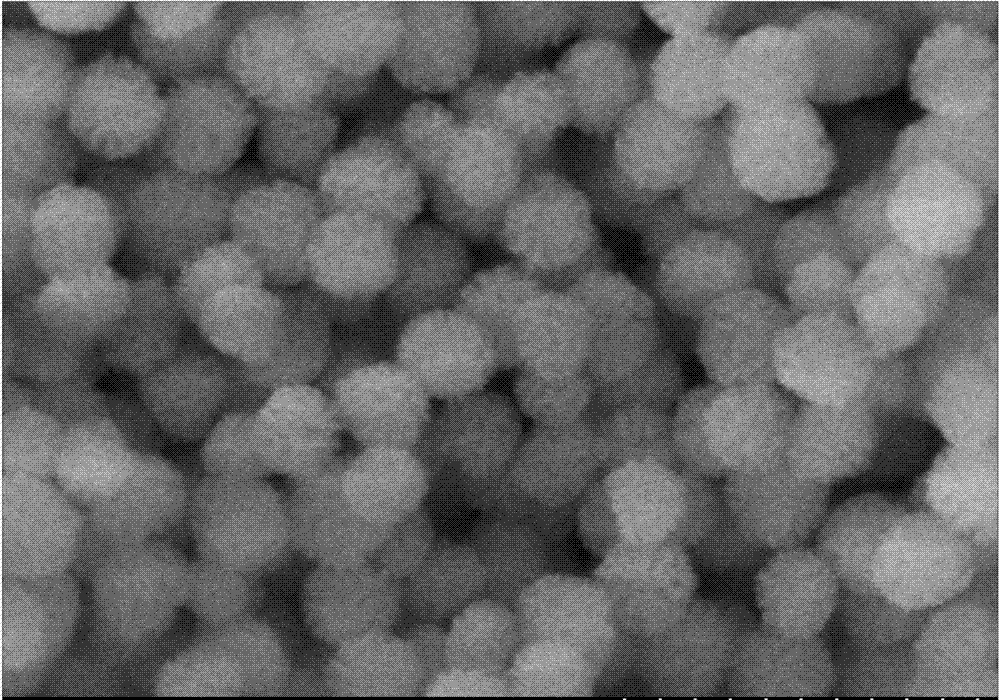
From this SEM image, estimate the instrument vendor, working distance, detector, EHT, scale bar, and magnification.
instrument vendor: Hitachi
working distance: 4 mm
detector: SE(U)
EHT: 3 kV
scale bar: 300 nm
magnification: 150 K X